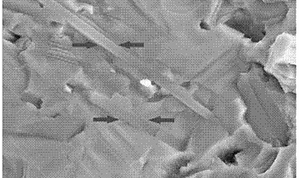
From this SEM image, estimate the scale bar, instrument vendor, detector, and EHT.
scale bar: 2000 nm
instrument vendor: Zeiss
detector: SE2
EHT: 15 kV